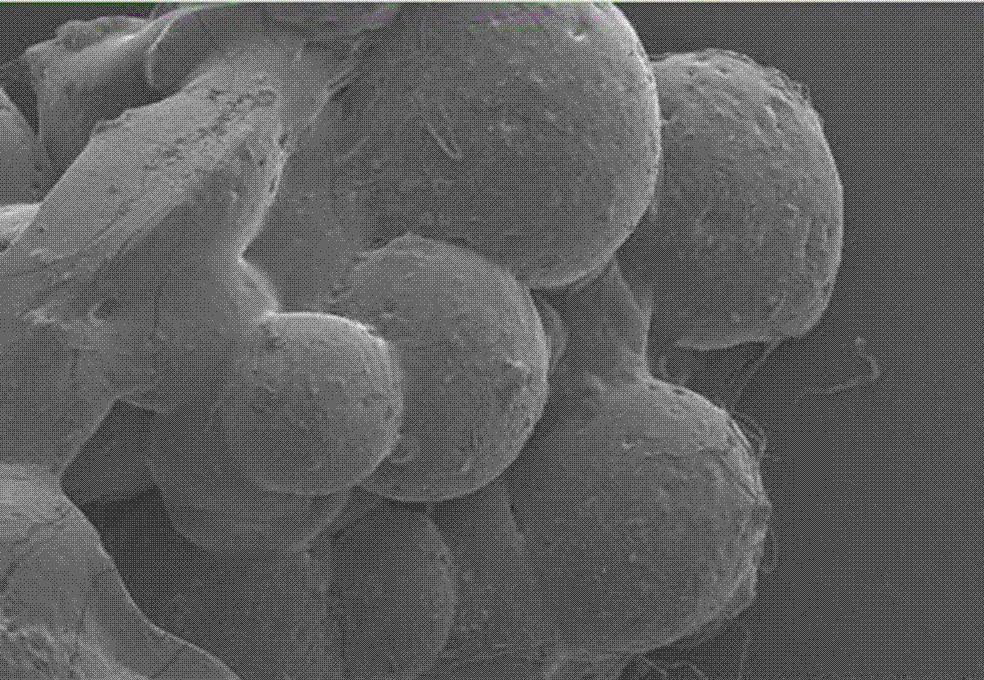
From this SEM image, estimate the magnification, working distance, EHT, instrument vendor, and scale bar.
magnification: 0.04 K X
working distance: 11.5 mm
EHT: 5 kV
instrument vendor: Hitachi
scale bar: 1000000 nm